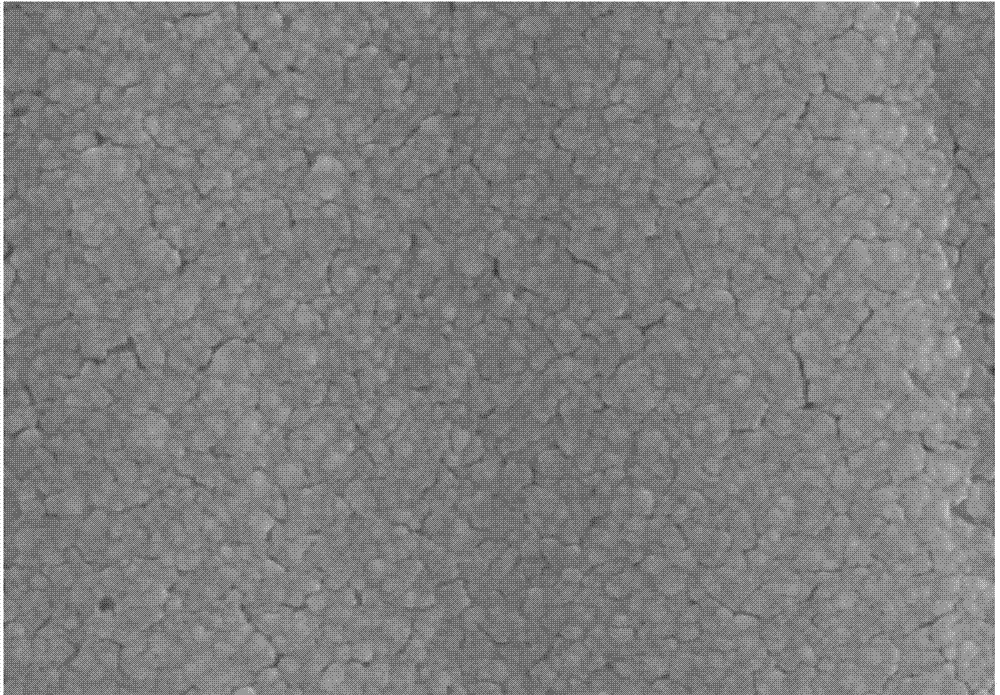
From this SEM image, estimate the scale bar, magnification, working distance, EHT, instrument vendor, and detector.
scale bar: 1000 nm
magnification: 50 K X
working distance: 11.8 mm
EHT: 20 kV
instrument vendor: Hitachi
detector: SE(M)